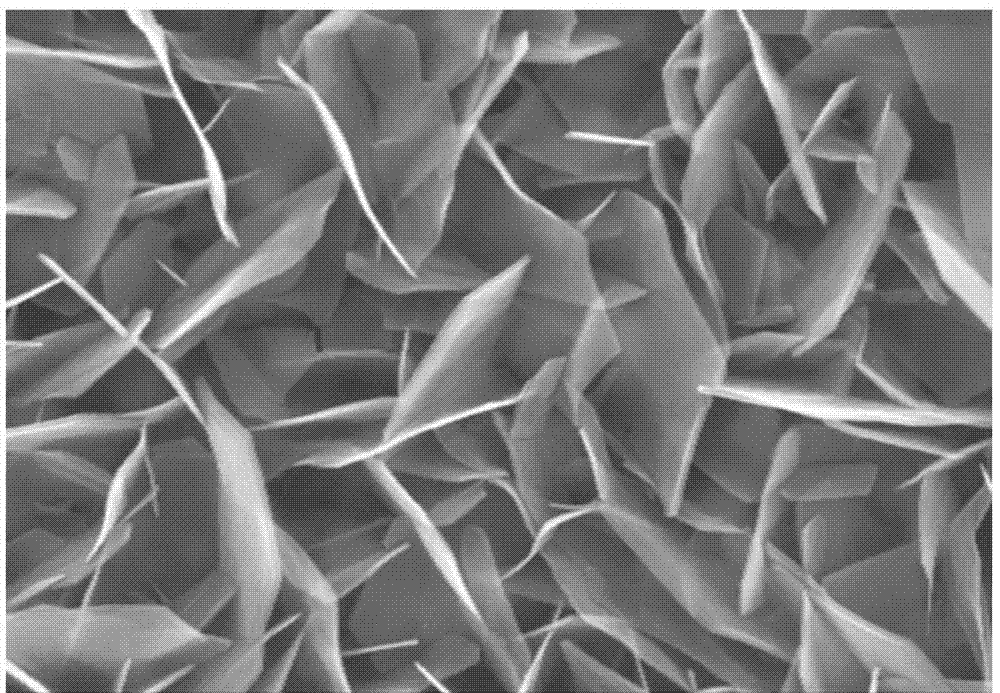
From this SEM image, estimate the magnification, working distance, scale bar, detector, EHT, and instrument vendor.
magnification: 100 K X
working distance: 5.5 mm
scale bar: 200 nm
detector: InLens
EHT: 20 kV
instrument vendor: Zeiss